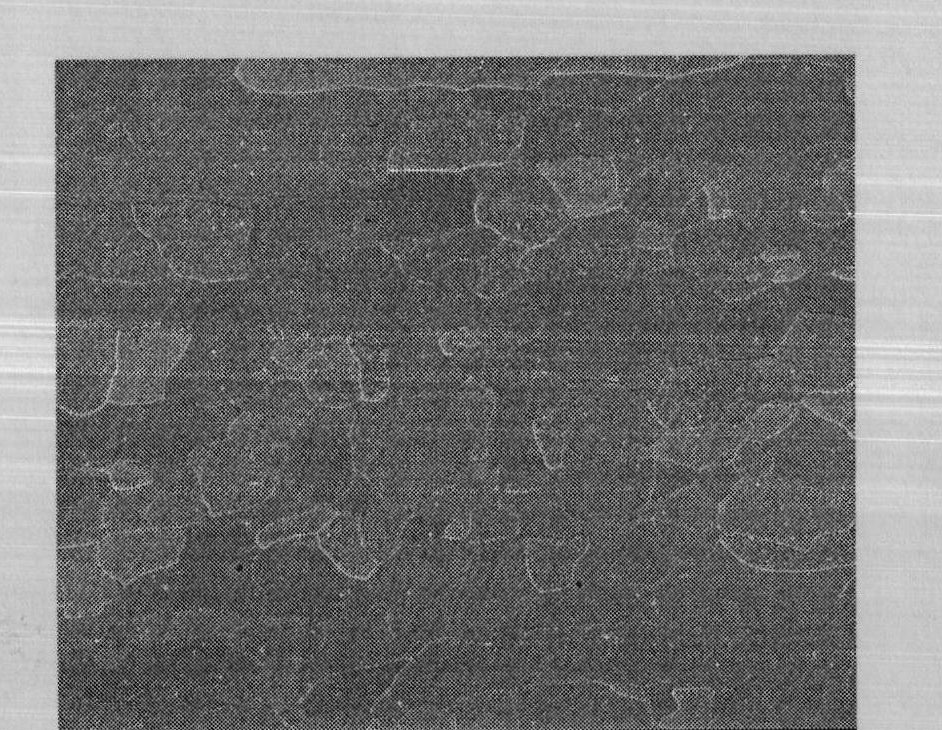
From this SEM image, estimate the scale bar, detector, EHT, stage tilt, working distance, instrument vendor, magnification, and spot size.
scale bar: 400000 nm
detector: ETD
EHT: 20 kV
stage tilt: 0°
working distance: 15.9 mm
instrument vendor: FEI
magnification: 0.3 K X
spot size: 4.5